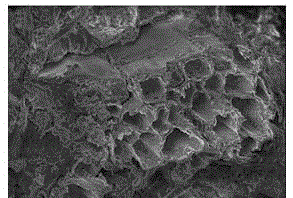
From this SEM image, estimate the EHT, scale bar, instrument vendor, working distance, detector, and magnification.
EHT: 5 kV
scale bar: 50000 nm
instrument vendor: Hitachi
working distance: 7.6 mm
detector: SE(M)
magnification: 1 K X